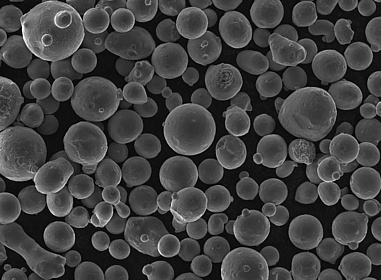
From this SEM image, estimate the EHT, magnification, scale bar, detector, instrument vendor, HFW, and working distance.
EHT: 15 kV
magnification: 1 K X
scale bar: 50000 nm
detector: SE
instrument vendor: Tescan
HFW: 276 µm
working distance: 8.01 mm